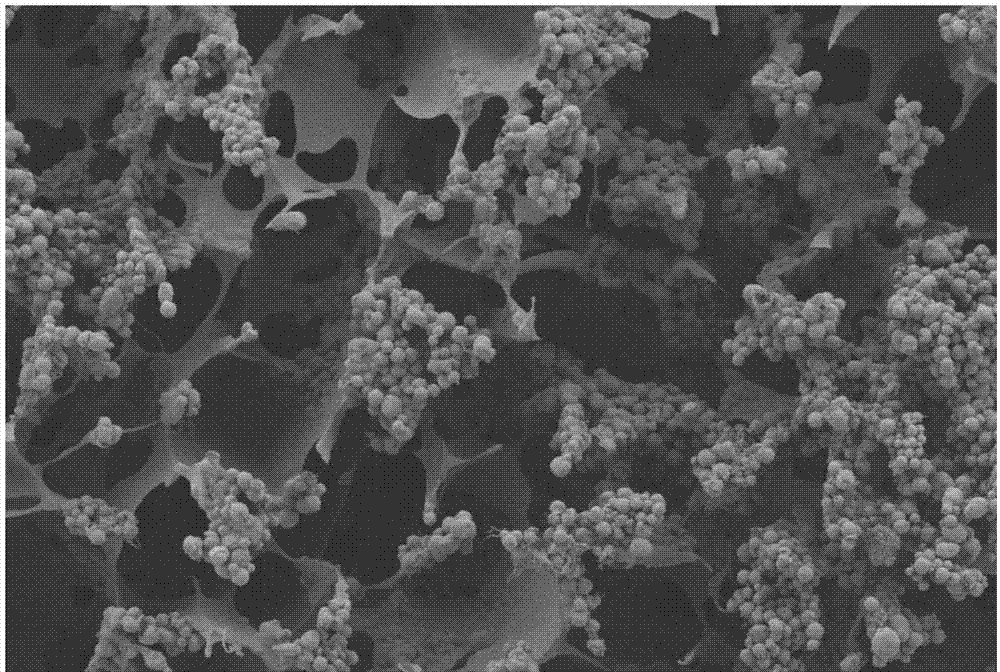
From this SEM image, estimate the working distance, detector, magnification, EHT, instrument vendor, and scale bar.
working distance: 6.7 mm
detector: SE2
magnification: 0.2 K X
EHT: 3 kV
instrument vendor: Zeiss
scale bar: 20000 nm